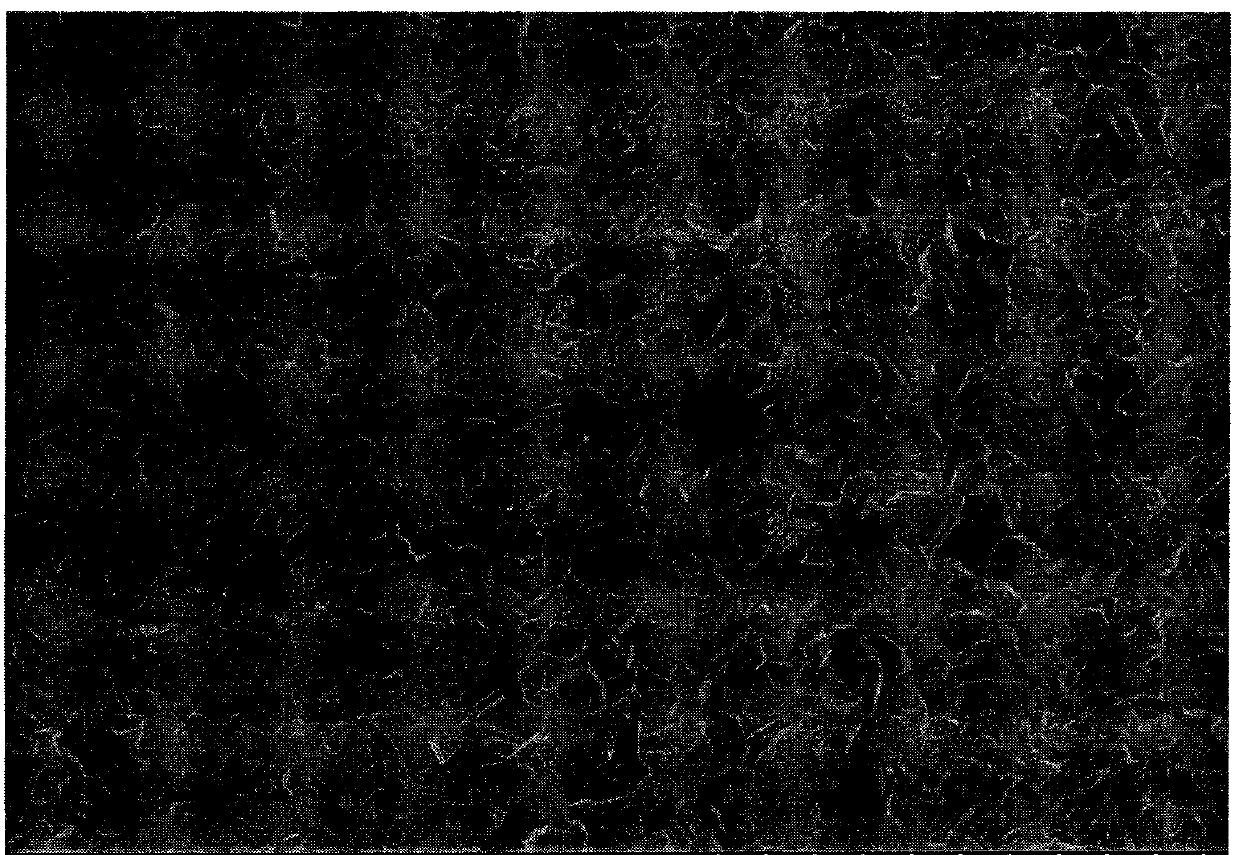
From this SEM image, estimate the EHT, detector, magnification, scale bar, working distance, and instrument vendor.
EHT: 5 kV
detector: SE(U)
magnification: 1 K X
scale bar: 50000 nm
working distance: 4.2 mm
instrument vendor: Hitachi